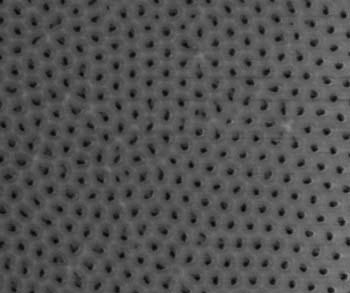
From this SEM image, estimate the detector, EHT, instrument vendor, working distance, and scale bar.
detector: ETD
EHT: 2 kV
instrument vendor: FEI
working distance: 7 mm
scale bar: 500 nm